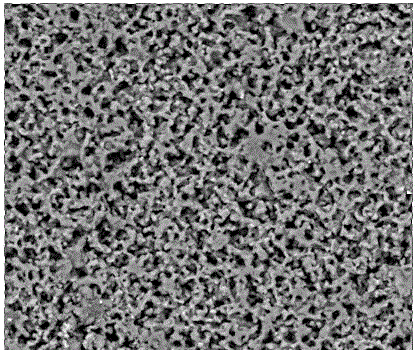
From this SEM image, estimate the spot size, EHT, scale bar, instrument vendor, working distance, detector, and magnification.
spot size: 5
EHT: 20 kV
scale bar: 50000 nm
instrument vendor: FEI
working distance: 10.3 mm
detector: BSED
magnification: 1 K X